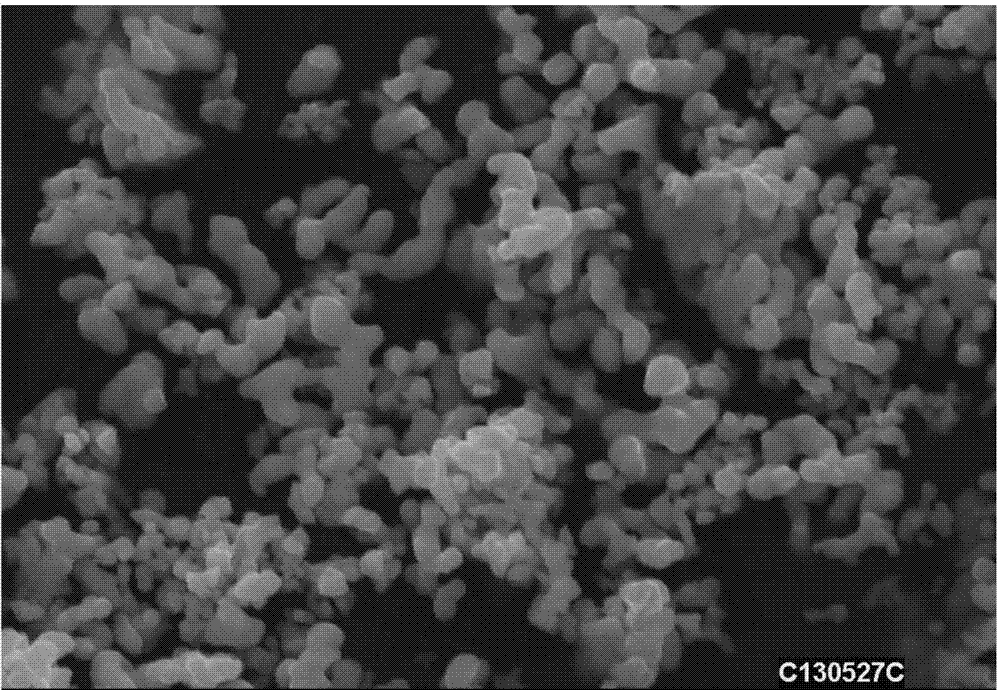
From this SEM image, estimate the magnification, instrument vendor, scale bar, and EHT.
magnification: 5 K X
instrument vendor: other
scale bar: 10000 nm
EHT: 25 kV